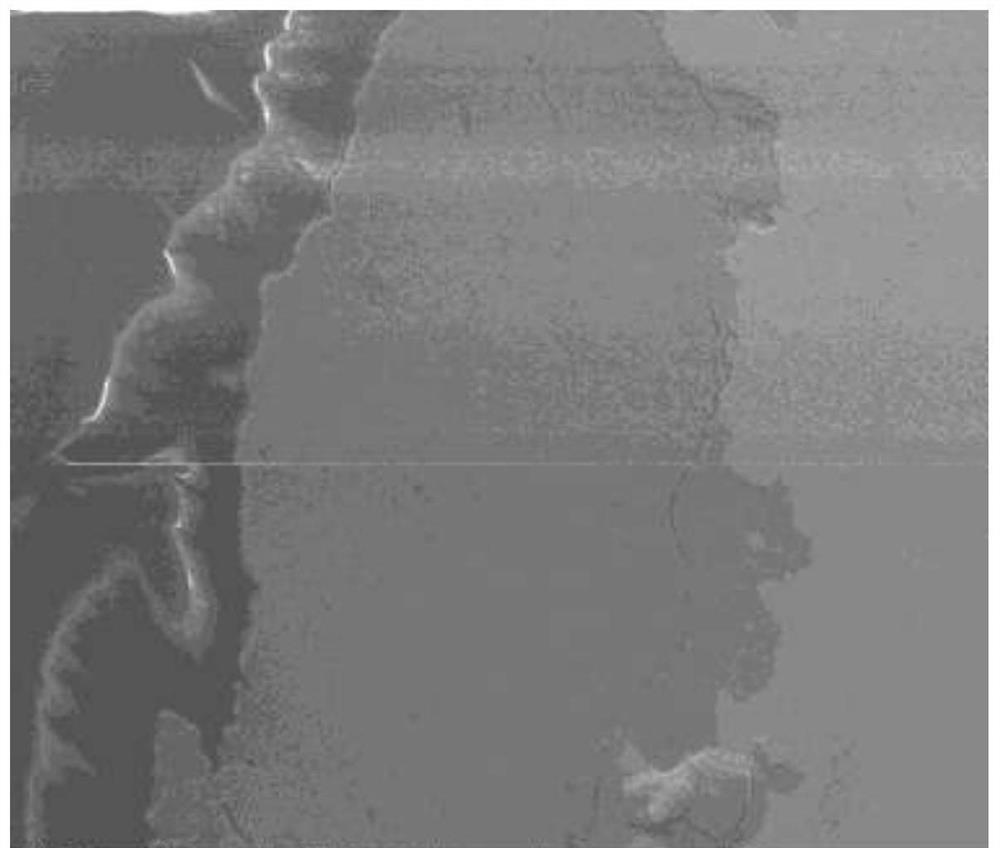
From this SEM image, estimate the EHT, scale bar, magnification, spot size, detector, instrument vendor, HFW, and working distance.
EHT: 20 kV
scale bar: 100000 nm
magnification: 0.8 K X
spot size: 3.5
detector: ETD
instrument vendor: FEI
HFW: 373 µm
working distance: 45.6 mm